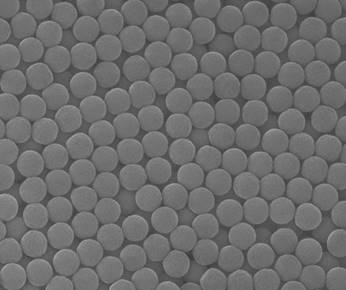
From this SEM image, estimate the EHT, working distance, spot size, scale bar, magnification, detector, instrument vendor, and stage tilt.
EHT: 20 kV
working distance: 8.8 mm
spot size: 3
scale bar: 2000 nm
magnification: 50 K X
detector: ETD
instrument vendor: FEI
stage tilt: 0°